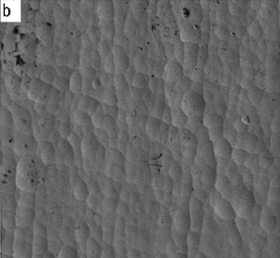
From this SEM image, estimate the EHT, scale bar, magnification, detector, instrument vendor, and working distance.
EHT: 5 kV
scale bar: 10000 nm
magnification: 0.5 K X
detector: LEI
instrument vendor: JEOL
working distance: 8.2 mm